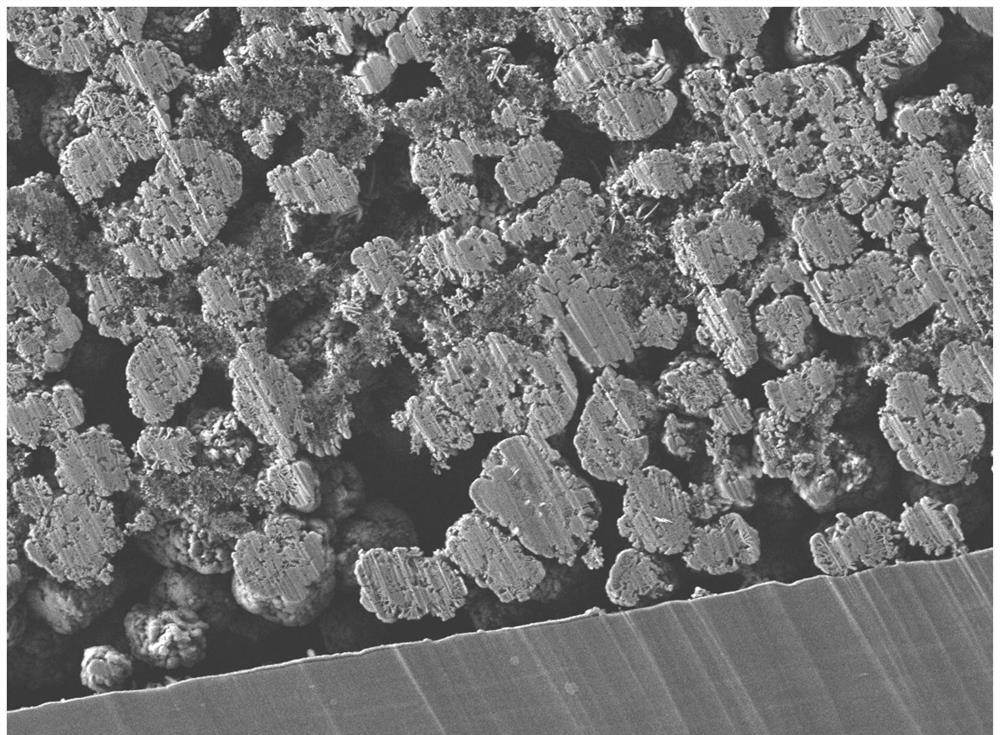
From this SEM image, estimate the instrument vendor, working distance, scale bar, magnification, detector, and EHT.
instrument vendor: JEOL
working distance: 8.7 mm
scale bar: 10000 nm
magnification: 2 K X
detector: LEI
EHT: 5 kV